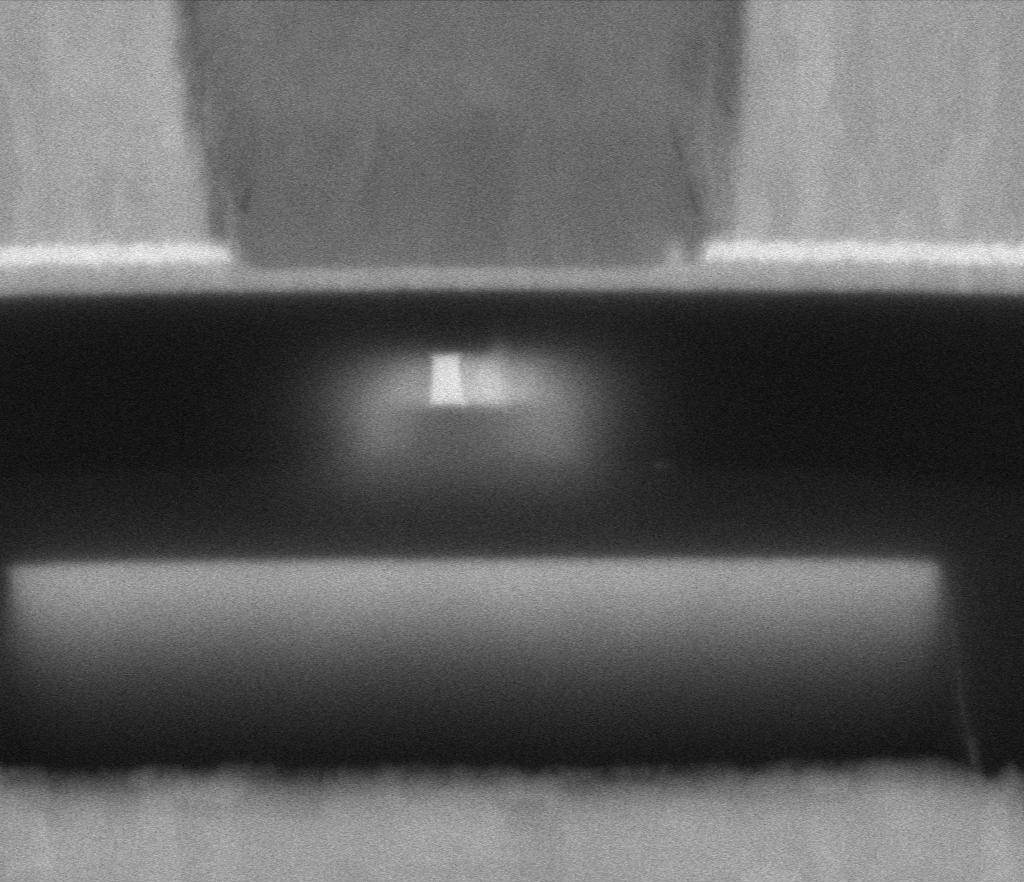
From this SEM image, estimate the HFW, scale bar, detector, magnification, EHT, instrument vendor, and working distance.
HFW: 0.635 µm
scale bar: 100 nm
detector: MD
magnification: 568.609 K X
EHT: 5 kV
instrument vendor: Thermo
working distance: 2 mm